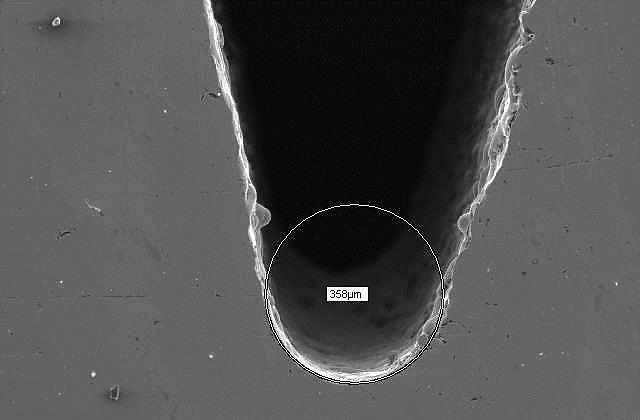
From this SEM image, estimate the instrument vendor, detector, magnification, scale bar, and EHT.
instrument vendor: JEOL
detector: SEI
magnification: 0.1 K X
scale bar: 100000 nm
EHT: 20 kV